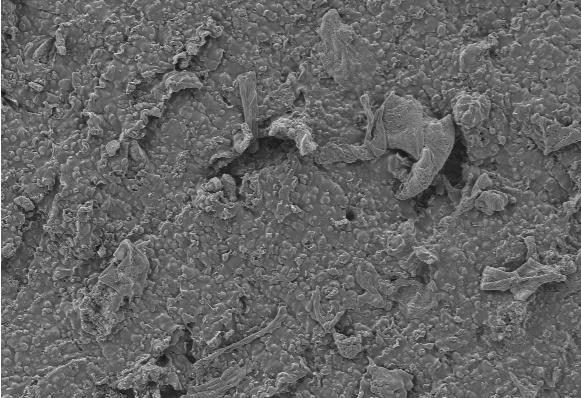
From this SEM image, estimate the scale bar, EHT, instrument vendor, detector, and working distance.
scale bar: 20000 nm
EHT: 5 kV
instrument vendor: Zeiss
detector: SE2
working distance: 5.7 mm